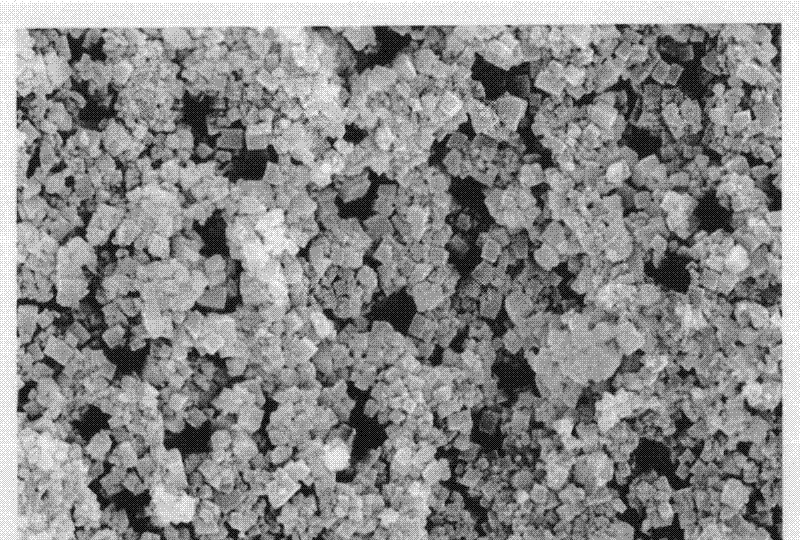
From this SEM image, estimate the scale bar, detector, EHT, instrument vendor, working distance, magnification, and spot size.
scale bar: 10000 nm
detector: SE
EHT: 20 kV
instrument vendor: FEI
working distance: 5.3 mm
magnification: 2 K X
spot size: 2.5